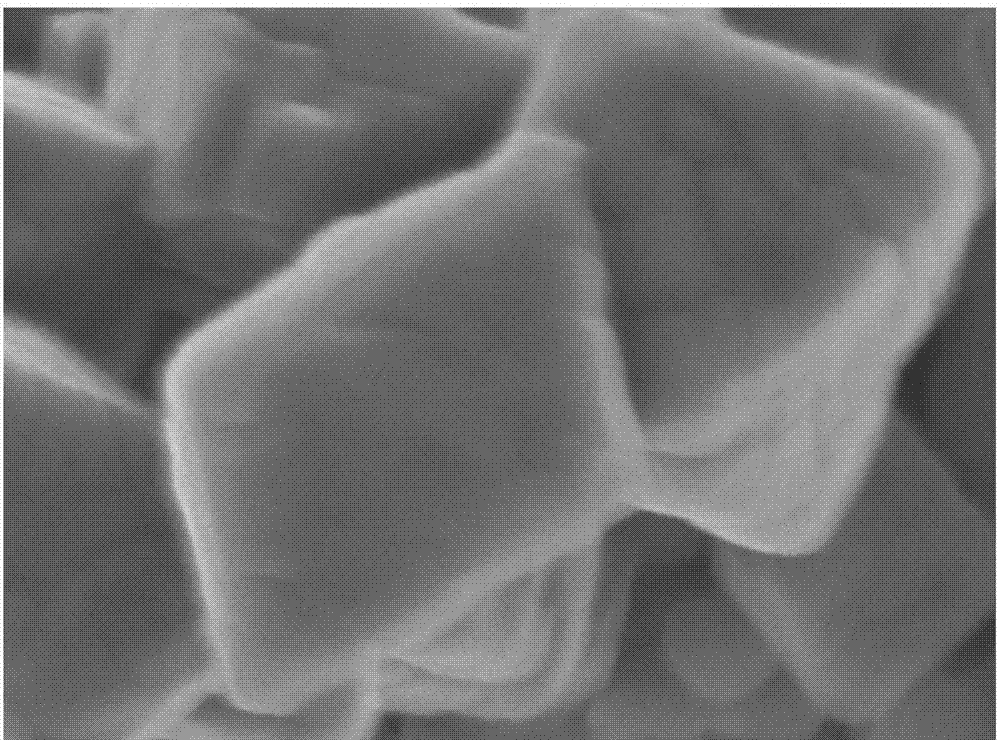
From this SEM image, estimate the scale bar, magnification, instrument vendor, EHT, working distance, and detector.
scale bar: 100 nm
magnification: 80 K X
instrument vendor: JEOL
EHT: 5 kV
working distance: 7.9 mm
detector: SEI